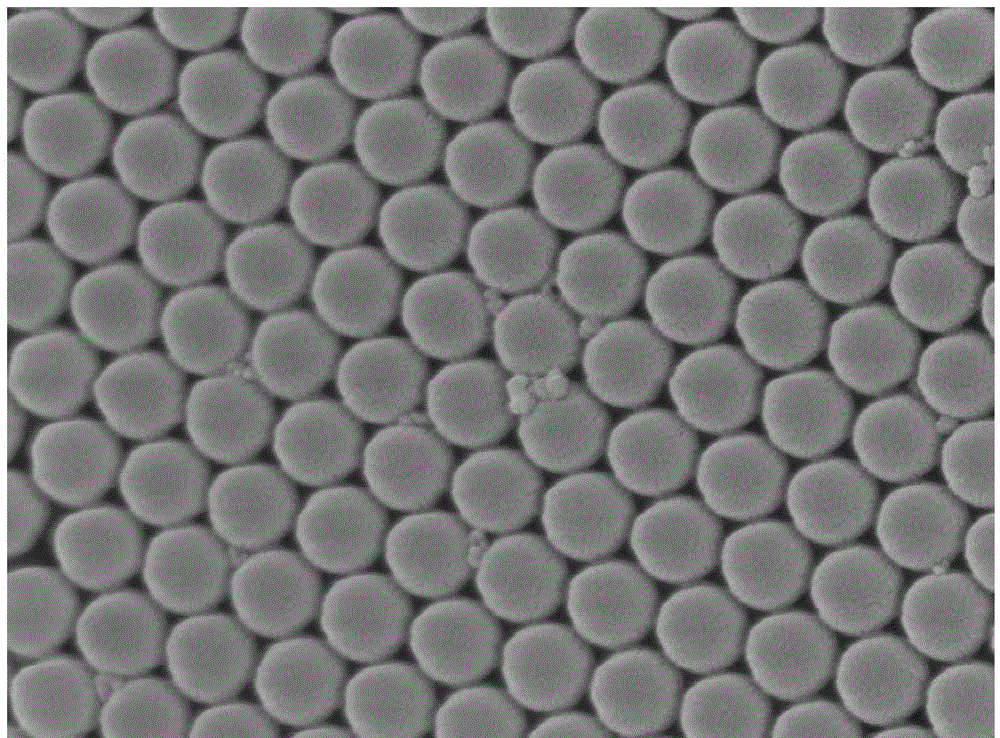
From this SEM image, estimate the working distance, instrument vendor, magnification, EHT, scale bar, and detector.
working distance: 6.5 mm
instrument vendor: JEOL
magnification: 50 K X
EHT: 5 kV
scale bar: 100 nm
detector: SEI